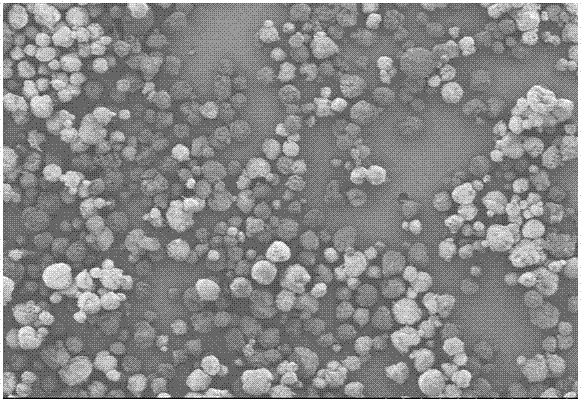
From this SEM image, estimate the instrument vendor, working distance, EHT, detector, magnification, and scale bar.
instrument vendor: Hitachi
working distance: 11 mm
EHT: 3 kV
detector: SE(M)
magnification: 0.04 K X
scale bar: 1000000 nm